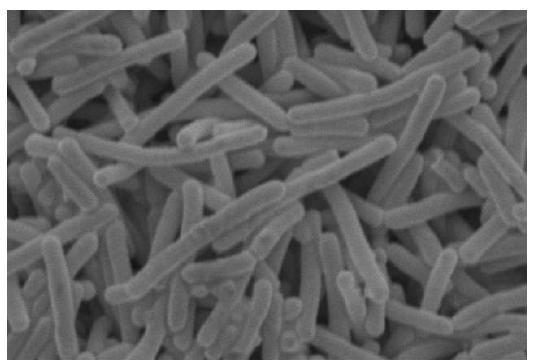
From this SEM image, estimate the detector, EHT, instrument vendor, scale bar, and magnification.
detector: SEI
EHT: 10 kV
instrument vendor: JEOL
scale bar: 1000 nm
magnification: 10 K X